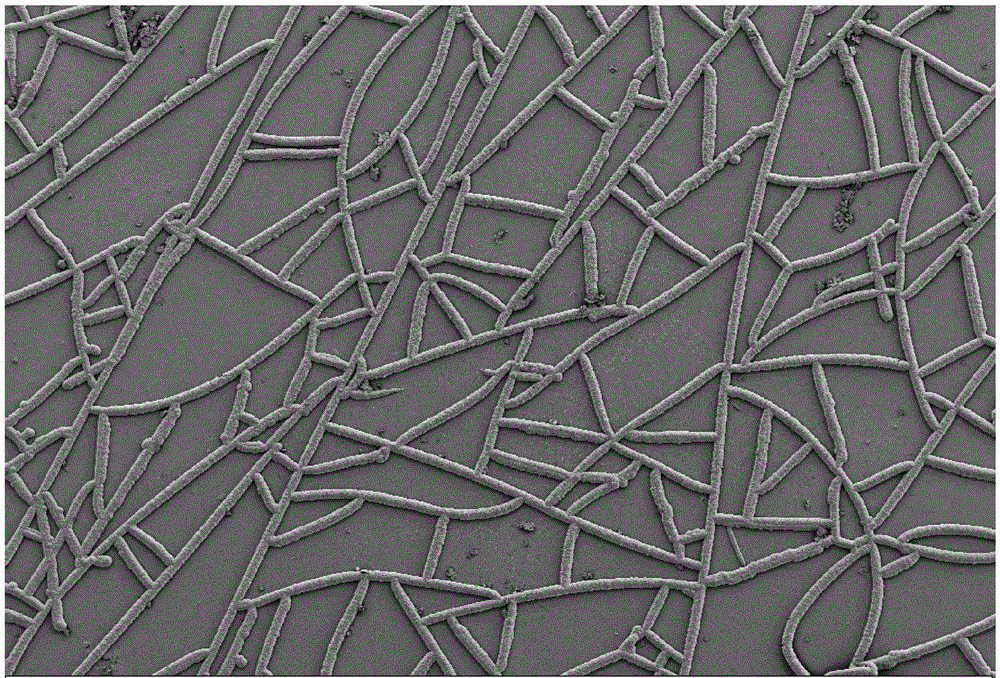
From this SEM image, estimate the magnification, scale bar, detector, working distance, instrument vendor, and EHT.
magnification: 0.3 K X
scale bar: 100000 nm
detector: SE2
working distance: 5 mm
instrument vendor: Zeiss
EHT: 5 kV